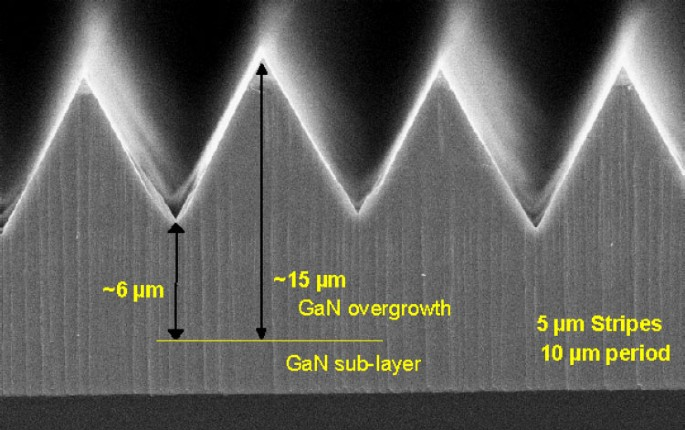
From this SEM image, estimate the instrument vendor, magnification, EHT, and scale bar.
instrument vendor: JEOL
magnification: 3 K X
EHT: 15 kV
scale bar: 10000 nm